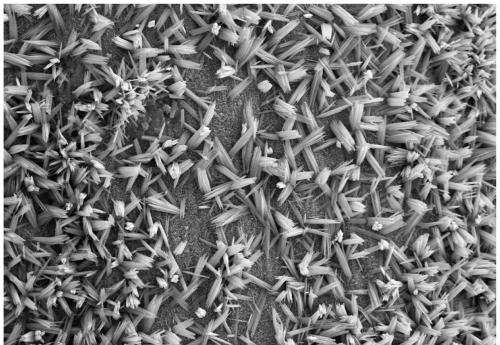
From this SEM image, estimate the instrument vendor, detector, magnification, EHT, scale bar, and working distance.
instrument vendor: Zeiss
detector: SE2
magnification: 5 K X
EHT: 15 kV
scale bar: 2000 nm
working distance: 12.8 mm